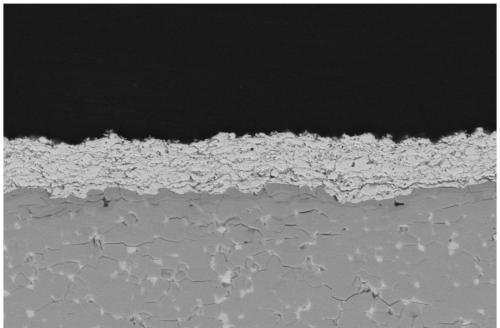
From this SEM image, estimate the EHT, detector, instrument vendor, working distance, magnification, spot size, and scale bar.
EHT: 20 kV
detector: BSED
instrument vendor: FEI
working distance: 12.3 mm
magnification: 3 K X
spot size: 3.5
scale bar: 30000 nm